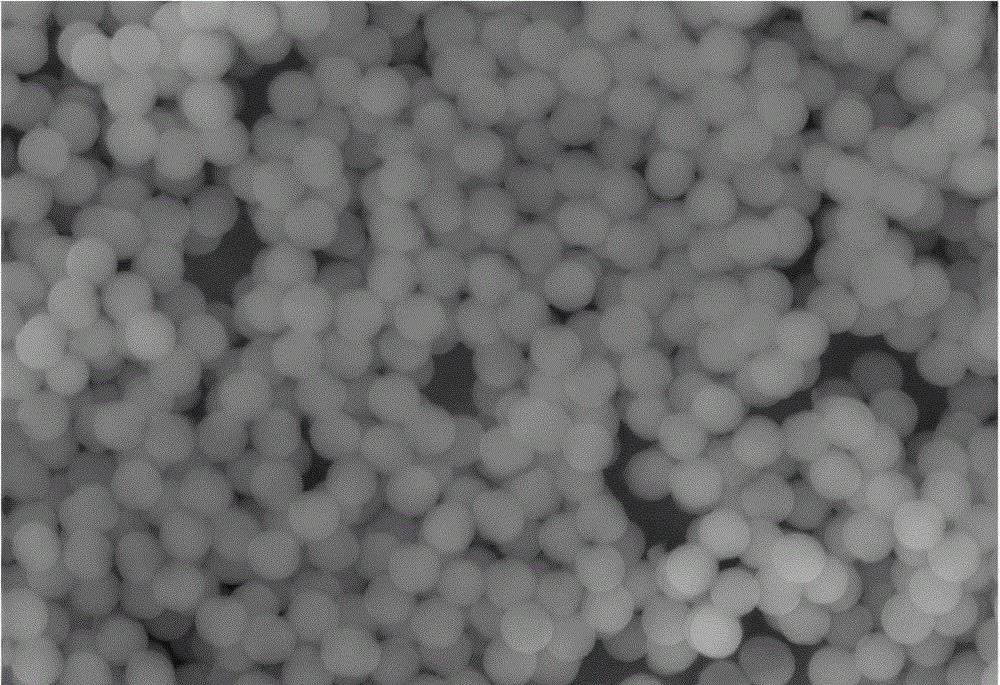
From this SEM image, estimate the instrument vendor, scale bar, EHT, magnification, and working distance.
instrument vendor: Tescan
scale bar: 2000 nm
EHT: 20 kV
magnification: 15 K X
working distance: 15.1 mm